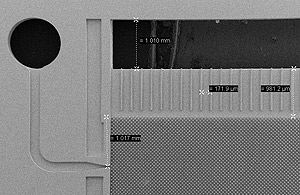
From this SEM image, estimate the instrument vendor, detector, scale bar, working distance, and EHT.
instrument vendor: Zeiss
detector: SE2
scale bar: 1000000 nm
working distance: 19 mm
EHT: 10 kV